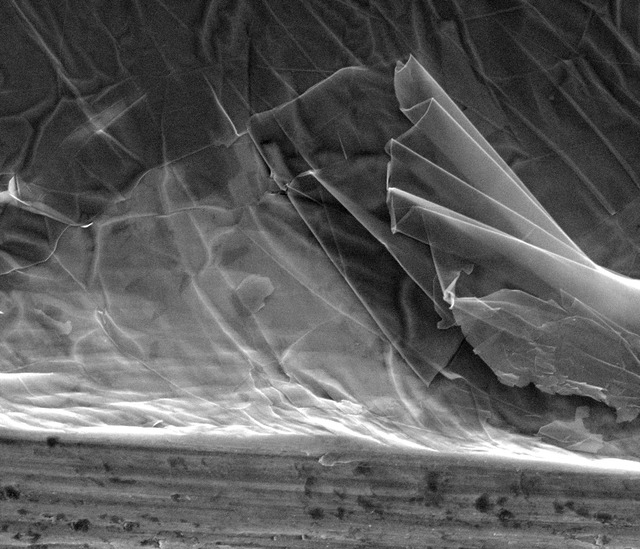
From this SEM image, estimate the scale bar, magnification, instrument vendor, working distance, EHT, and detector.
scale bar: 3000 nm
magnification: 12.496 K X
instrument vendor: FEI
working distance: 10.2 mm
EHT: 10 kV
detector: ETD